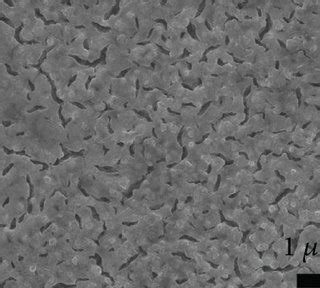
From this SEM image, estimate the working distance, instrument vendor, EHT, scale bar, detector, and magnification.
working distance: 10 mm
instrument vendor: JEOL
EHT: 15 kV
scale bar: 1000 nm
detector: LED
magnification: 10 K X